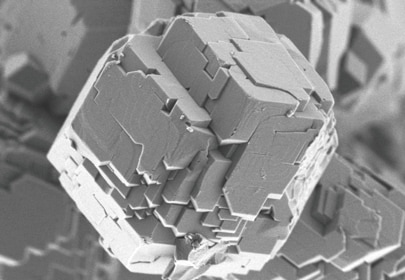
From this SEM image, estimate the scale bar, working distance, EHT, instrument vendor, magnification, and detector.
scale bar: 2000 nm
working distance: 2.1 mm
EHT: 0.8 kV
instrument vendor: Hitachi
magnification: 25 K X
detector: UD